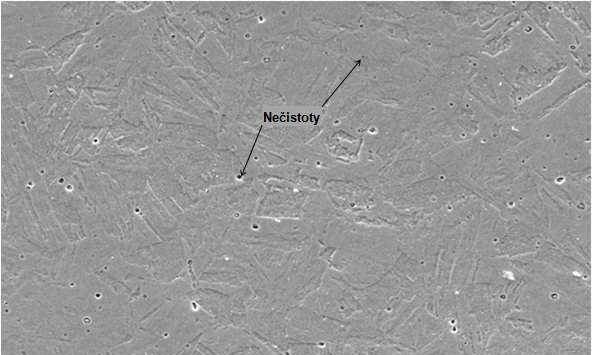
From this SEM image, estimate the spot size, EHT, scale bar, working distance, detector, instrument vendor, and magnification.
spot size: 4.5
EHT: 20 kV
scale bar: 50000 nm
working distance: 13.4 mm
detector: SE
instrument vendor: FEI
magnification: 0.5 K X